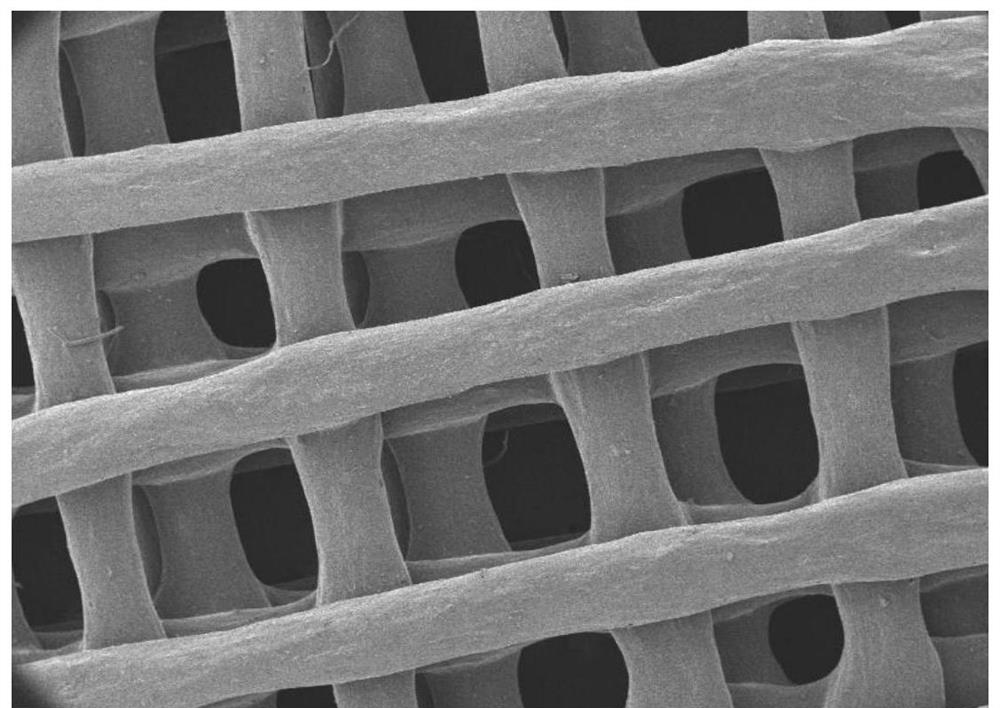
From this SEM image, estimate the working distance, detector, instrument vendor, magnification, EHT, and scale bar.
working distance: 12.3 mm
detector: SED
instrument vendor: JEOL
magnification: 0.03 K X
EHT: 5 kV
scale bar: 500000 nm